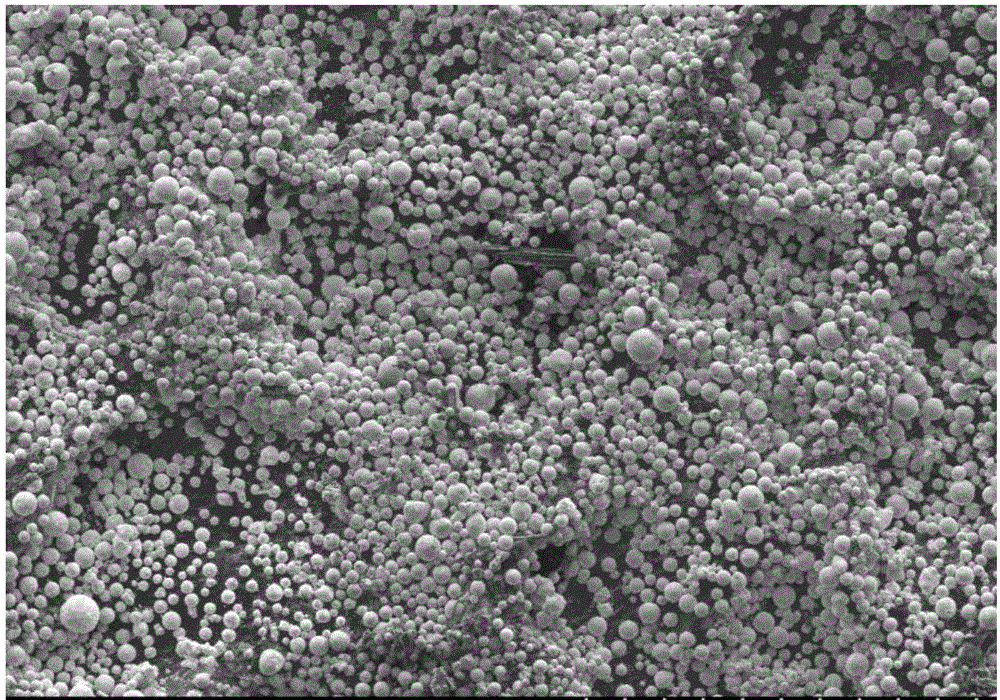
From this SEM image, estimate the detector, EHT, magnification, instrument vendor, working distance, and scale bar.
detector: SE(M)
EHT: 10 kV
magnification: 0.1 K X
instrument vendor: Hitachi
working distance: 12 mm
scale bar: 500000 nm